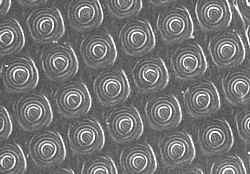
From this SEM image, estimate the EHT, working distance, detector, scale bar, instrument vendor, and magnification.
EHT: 3 kV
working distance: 15 mm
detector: SEI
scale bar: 1000 nm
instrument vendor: JEOL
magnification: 3 K X